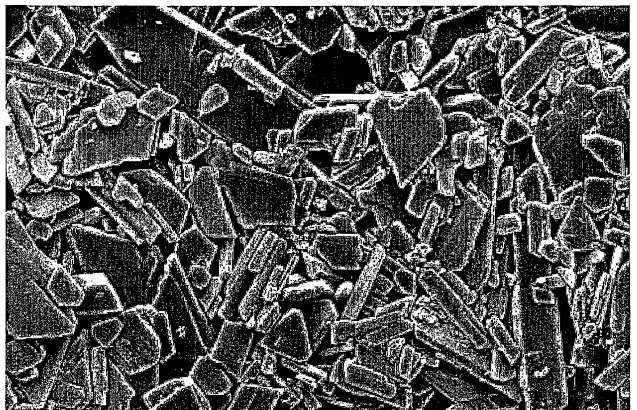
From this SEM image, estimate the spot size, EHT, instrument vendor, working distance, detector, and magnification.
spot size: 2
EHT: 3 kV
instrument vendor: FEI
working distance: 10 mm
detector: SE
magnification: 10 K X